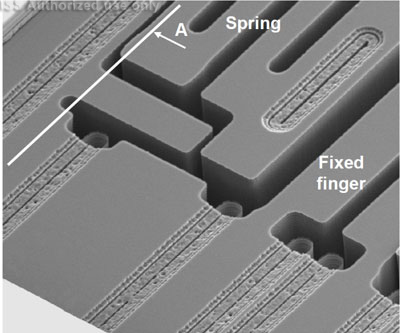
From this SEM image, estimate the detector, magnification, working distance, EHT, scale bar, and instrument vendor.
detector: ETD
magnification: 3.5 K X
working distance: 4.4 mm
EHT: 5 kV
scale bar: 10000 nm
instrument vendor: FEI